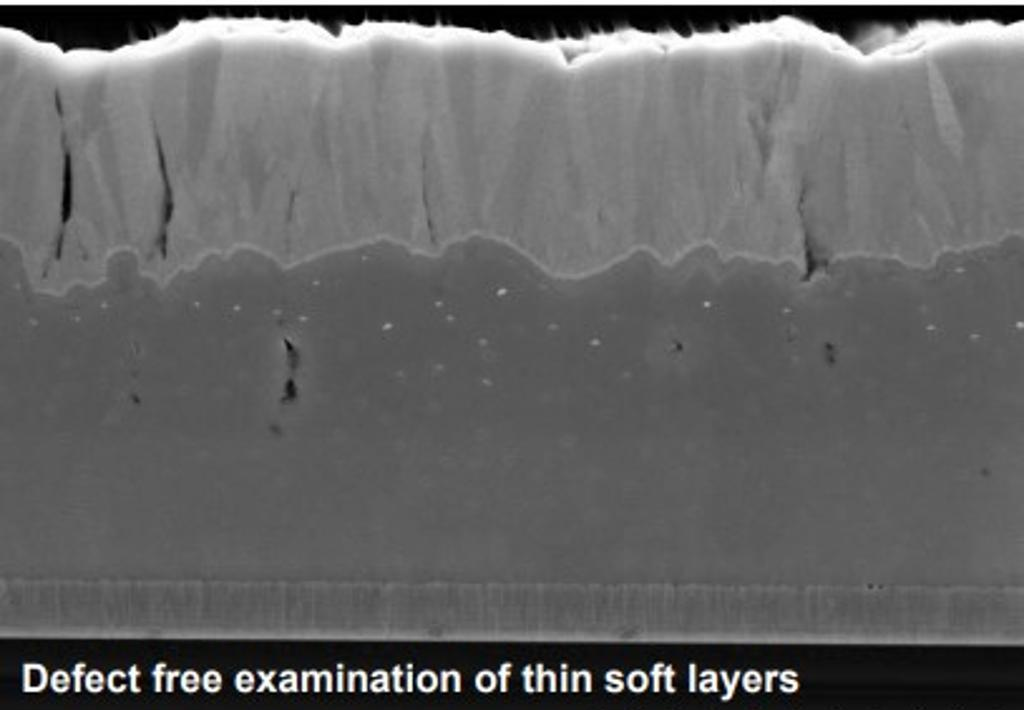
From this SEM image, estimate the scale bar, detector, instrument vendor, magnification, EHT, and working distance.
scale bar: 2000 nm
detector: SE(U,LA0)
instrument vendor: Hitachi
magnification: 25 K X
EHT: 5 kV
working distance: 1.8 mm